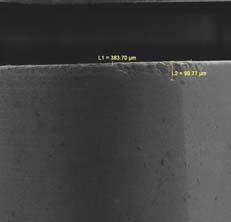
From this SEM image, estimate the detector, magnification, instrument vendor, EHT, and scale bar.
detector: SE Detector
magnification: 0.193 K X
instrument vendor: Tescan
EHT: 5 kV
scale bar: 500000 nm